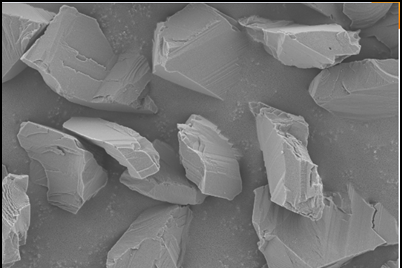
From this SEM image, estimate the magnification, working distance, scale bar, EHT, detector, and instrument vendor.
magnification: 5 K X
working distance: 7.8 mm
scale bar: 2000 nm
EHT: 3 kV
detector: SE2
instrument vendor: Zeiss